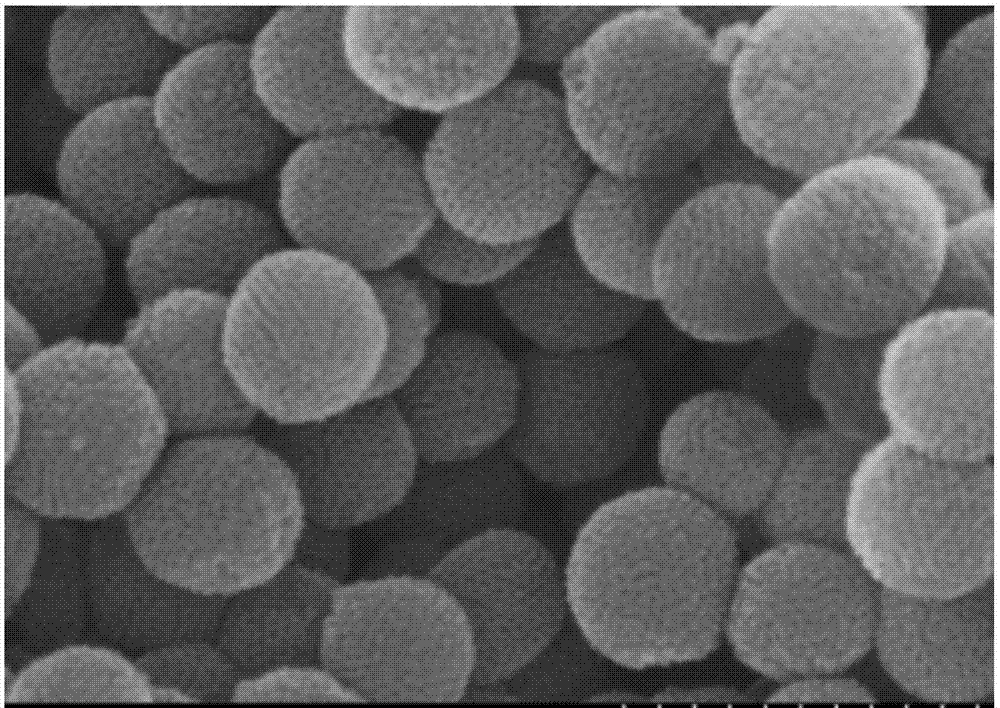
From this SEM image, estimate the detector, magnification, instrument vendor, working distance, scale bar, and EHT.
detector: SE(M)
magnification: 150 K X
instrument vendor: Hitachi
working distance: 8.5 mm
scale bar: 300 nm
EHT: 10 kV